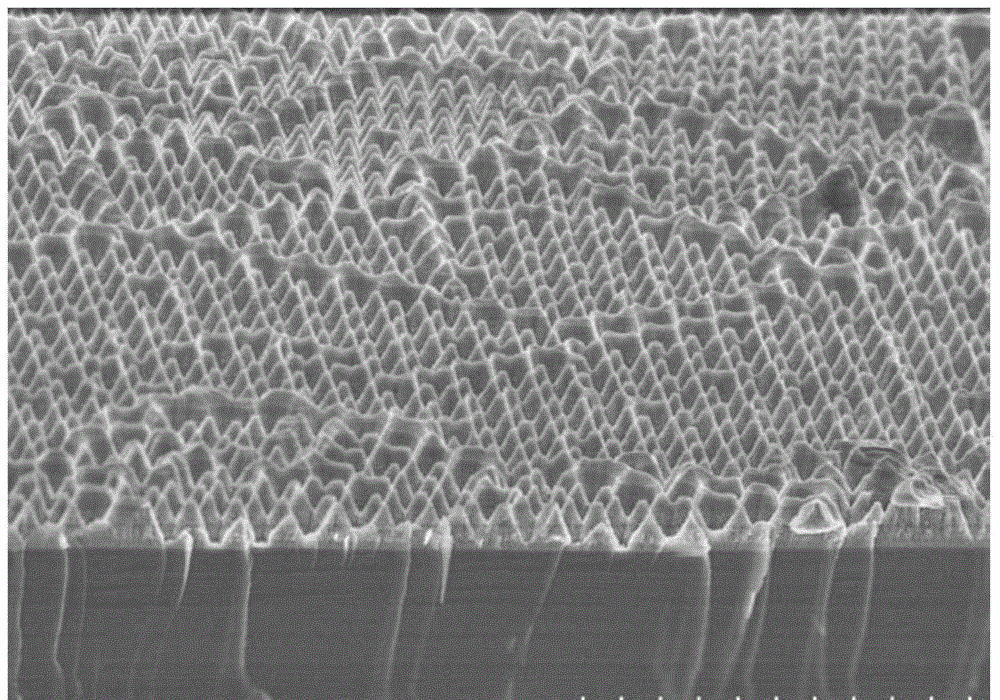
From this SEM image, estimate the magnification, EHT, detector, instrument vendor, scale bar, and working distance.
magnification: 10 K X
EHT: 3 kV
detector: SE(U)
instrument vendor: Hitachi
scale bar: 5000 nm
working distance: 6.2 mm